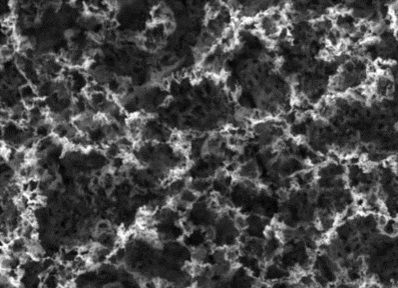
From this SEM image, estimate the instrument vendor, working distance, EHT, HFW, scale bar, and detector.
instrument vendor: Tescan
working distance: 1.77 mm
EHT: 5 kV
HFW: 5 µm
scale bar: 1000 nm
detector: SE